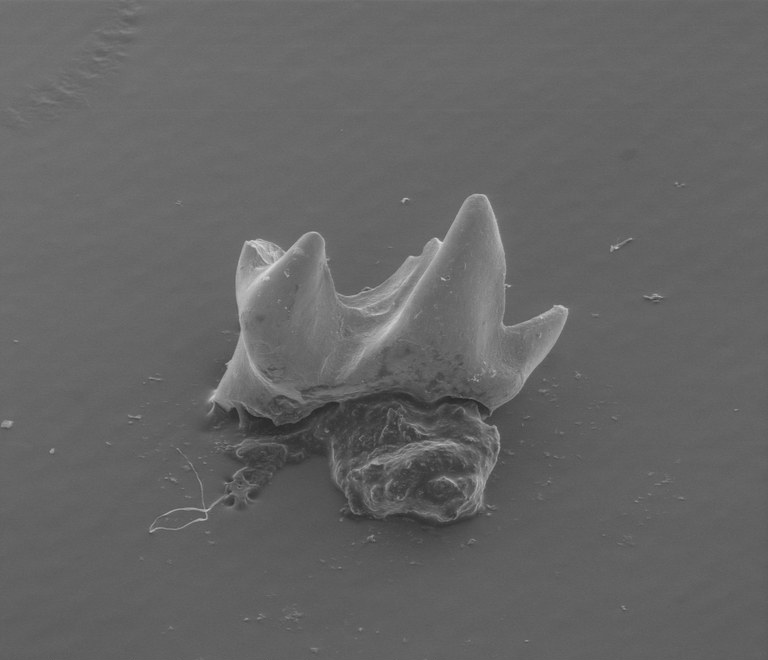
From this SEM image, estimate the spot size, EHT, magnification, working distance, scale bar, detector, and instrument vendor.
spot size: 4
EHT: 20 kV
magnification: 0.09 K X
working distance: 40.7 mm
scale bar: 1000000 nm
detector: LFD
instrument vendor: FEI